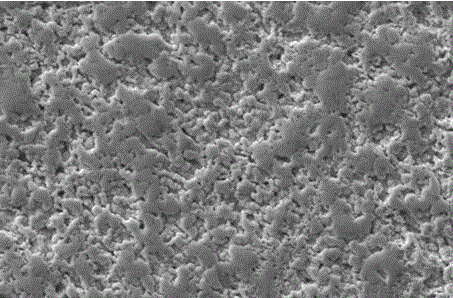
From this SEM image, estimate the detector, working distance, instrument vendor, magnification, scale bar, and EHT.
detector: SE1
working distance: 8.5 mm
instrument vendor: Zeiss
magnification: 1 K X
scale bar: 10000 nm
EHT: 20 kV